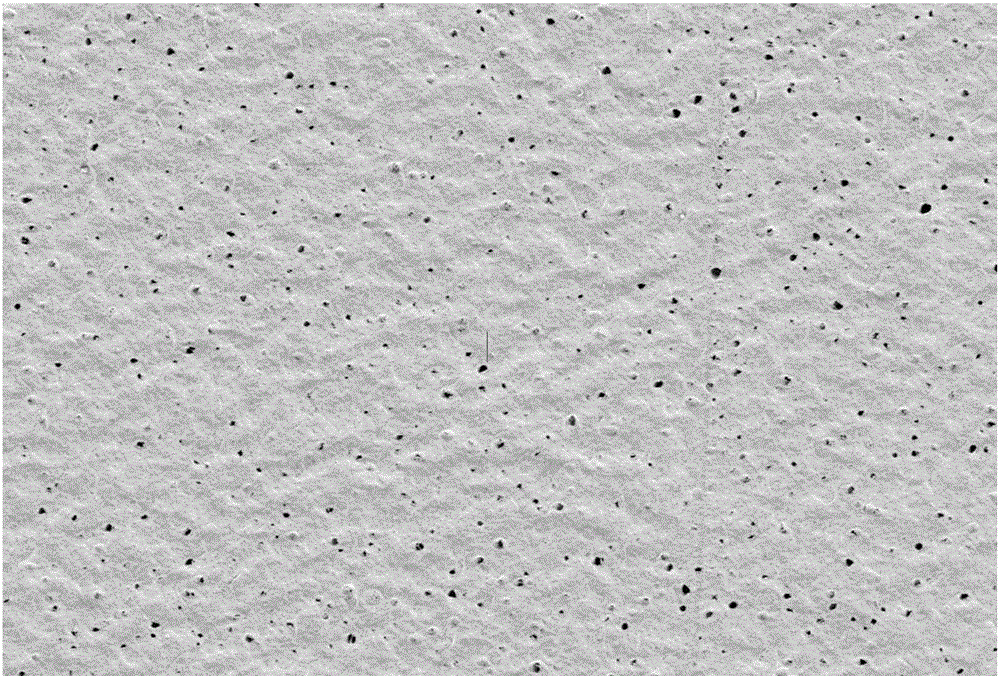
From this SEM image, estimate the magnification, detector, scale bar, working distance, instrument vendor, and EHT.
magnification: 5 K X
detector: SE2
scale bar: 2000 nm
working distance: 5.1 mm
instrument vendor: Zeiss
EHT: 5 kV